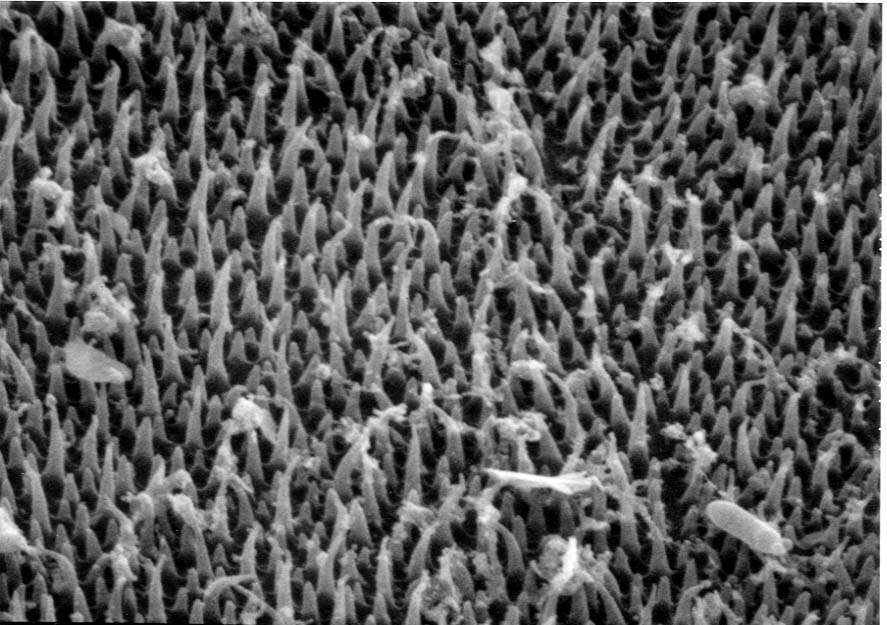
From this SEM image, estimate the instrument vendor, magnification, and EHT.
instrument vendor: JEOL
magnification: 10 K X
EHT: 20 kV